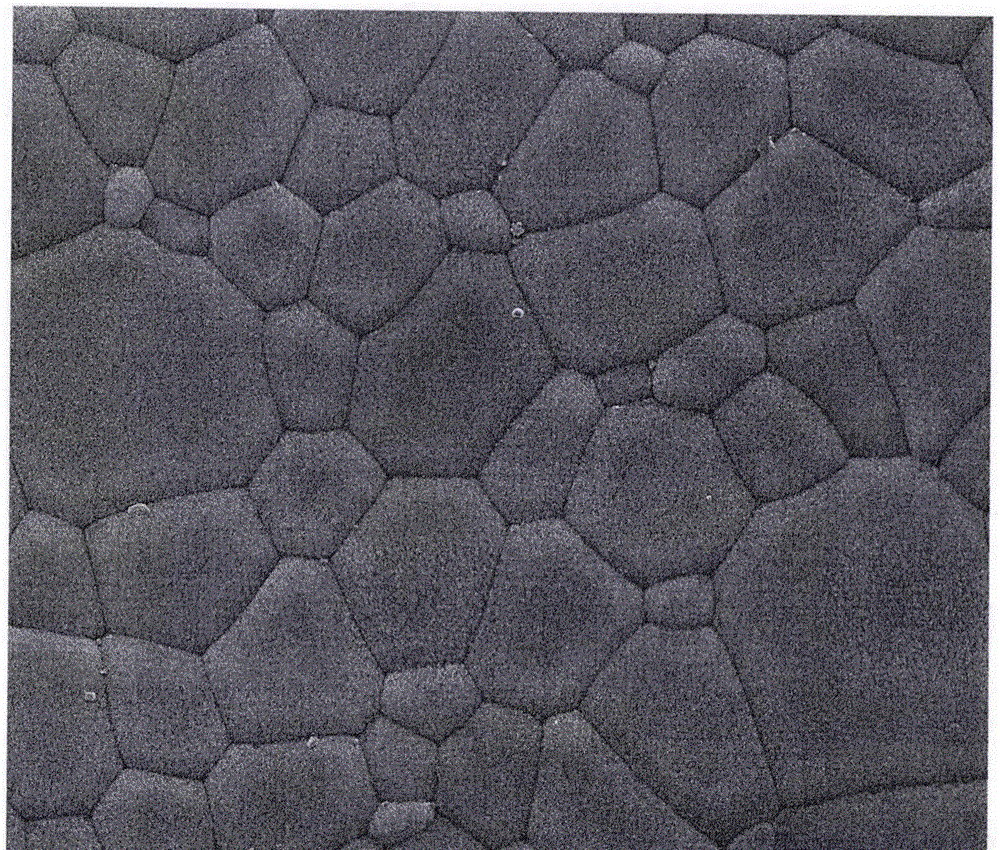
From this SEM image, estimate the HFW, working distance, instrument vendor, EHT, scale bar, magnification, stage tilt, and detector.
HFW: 29.8 µm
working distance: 10.3 mm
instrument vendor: FEI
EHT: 20 kV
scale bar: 10000 nm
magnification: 10 K X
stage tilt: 0°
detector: ETD